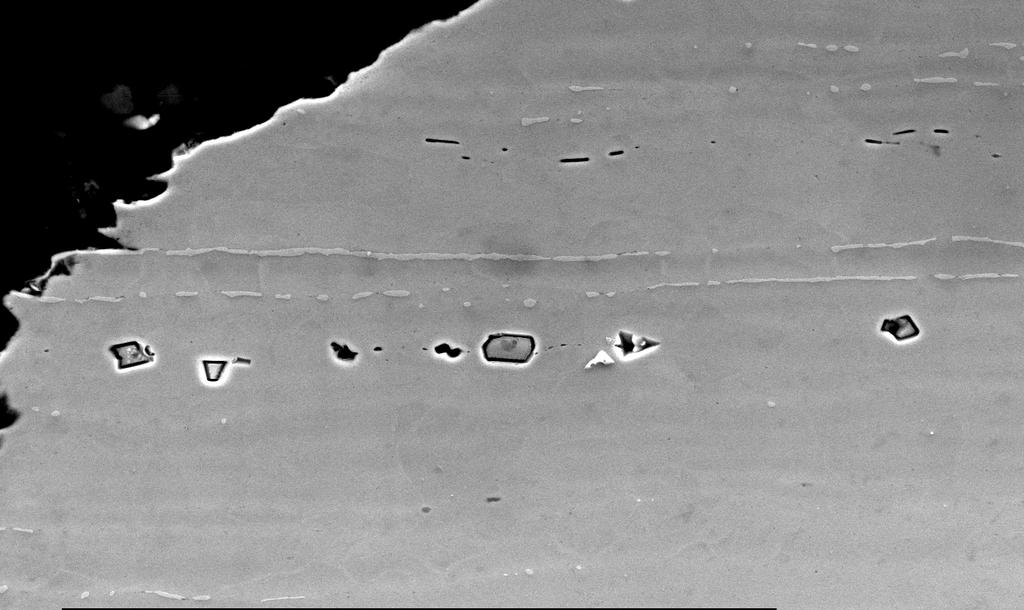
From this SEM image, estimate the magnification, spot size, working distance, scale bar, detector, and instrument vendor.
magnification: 0.8 K X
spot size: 4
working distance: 10.8 mm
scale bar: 20000 nm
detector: SE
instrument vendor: FEI